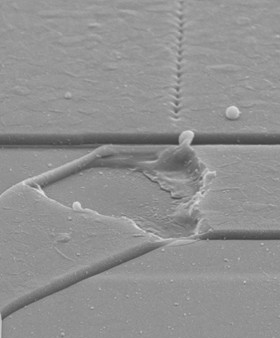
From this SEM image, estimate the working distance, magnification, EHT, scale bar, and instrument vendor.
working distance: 15 mm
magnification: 2.5 K X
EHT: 20 kV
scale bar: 8000 nm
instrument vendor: Zeiss